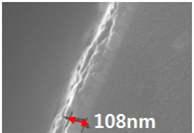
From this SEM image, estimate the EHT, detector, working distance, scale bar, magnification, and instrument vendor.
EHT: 10 kV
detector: SE(U)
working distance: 12.9 mm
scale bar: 500 nm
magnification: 100 K X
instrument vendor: Hitachi